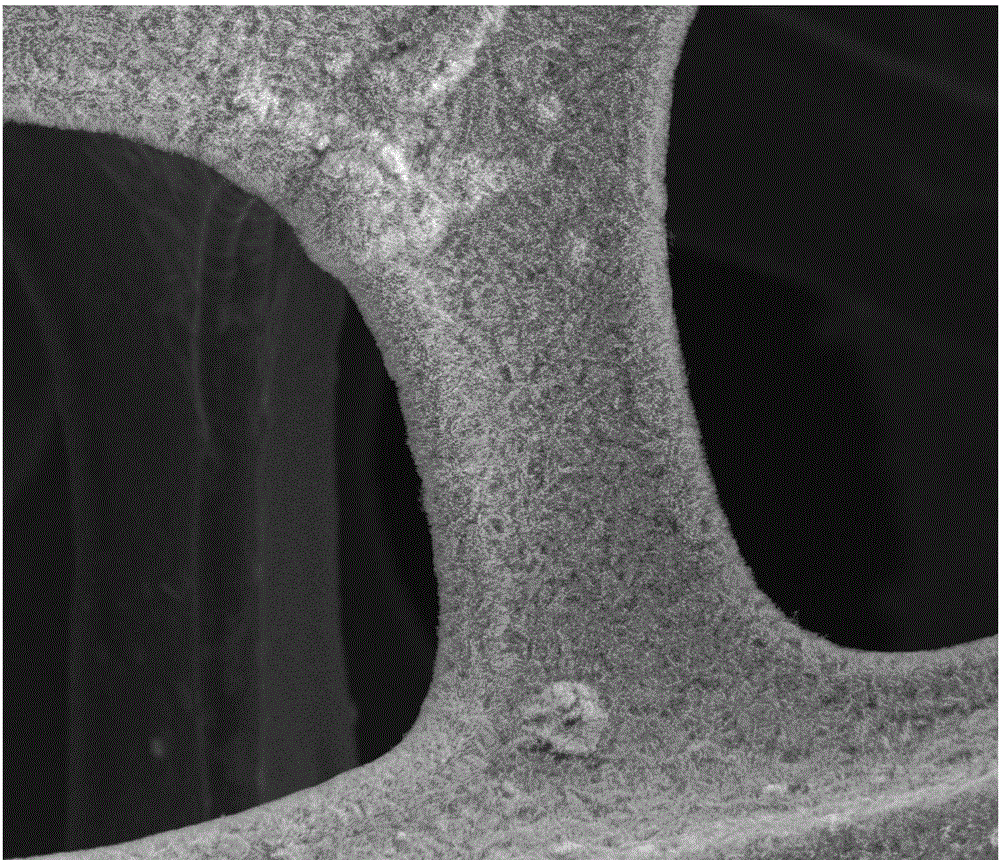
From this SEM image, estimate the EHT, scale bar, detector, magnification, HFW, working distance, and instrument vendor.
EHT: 30 kV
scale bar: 50000 nm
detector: SE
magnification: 1.5 K X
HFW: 199 µm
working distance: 9.9 mm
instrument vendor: FEI